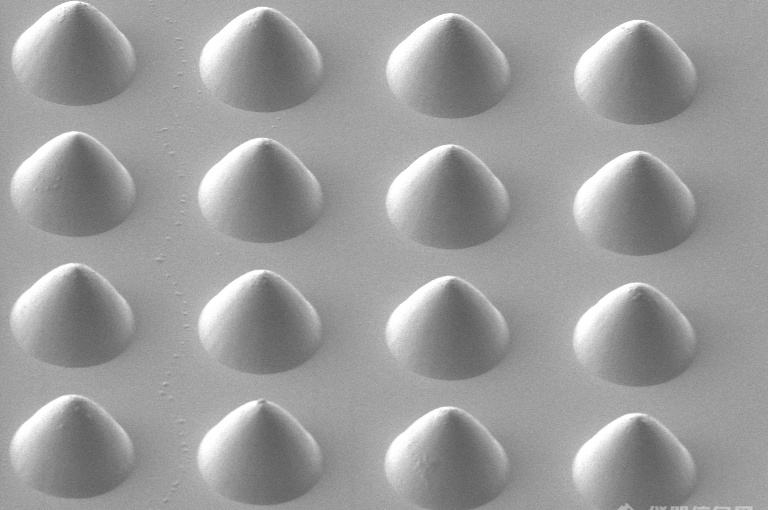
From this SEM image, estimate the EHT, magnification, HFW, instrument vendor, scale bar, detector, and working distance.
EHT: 2 kV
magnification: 2 K X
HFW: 104 µm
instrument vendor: FEI/Thermo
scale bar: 20000 nm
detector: ICE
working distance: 3.8 mm